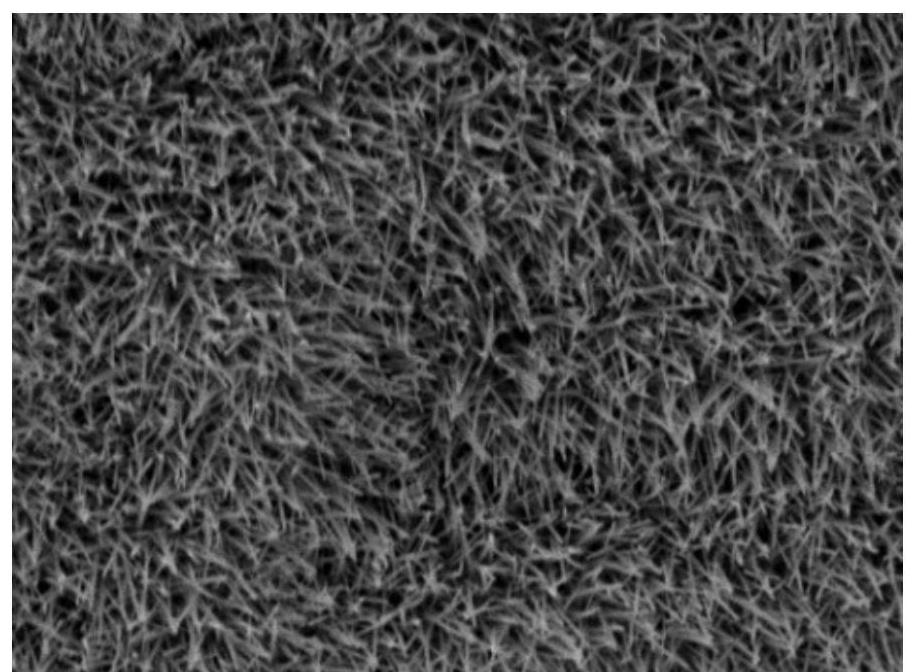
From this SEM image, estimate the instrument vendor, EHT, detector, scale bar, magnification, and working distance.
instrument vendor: JEOL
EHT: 10 kV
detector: SED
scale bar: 5000 nm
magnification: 3 K X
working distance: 10.8 mm